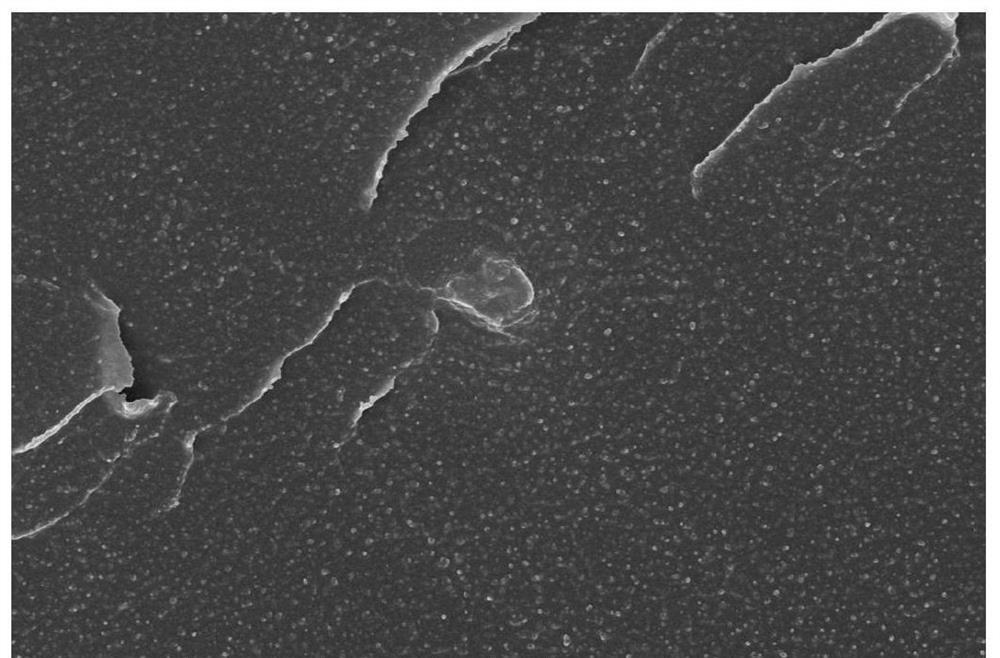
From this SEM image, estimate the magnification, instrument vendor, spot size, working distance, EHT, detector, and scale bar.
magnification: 40 K X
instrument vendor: FEI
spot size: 3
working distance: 6.9 mm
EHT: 20 kV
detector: SE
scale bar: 4000 nm